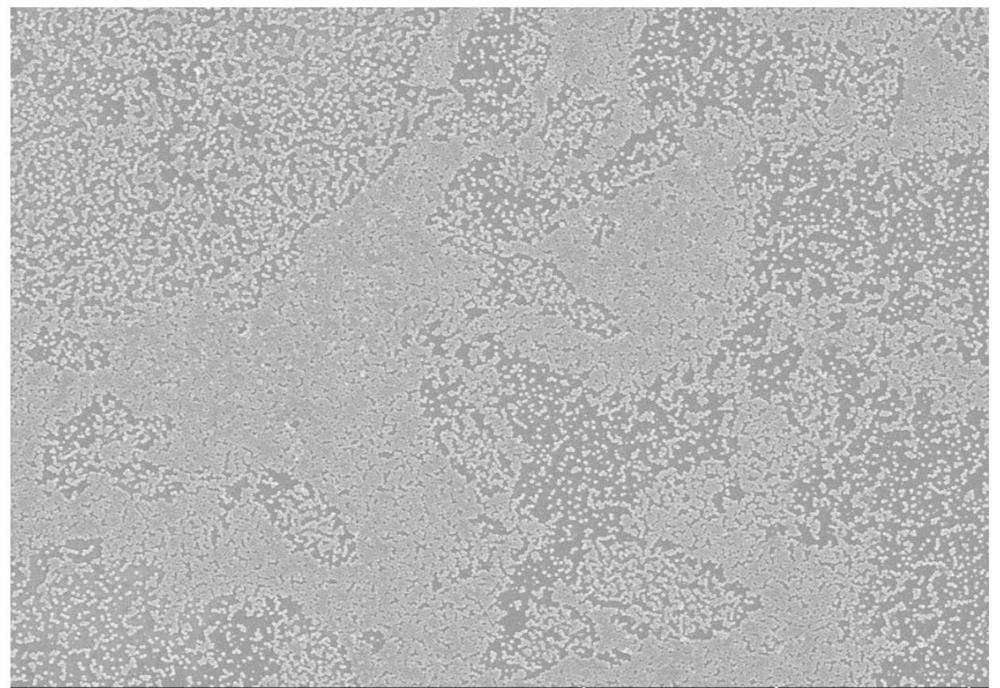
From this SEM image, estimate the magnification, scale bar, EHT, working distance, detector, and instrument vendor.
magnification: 5 K X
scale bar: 10000 nm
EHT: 3 kV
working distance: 8.4 mm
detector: SE(UL)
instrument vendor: Hitachi